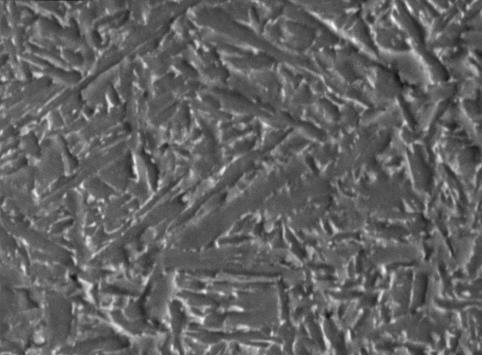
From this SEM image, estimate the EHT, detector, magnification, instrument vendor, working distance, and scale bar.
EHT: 20 kV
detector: SEI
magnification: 3 K X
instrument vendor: JEOL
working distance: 20.39 mm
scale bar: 5000 nm